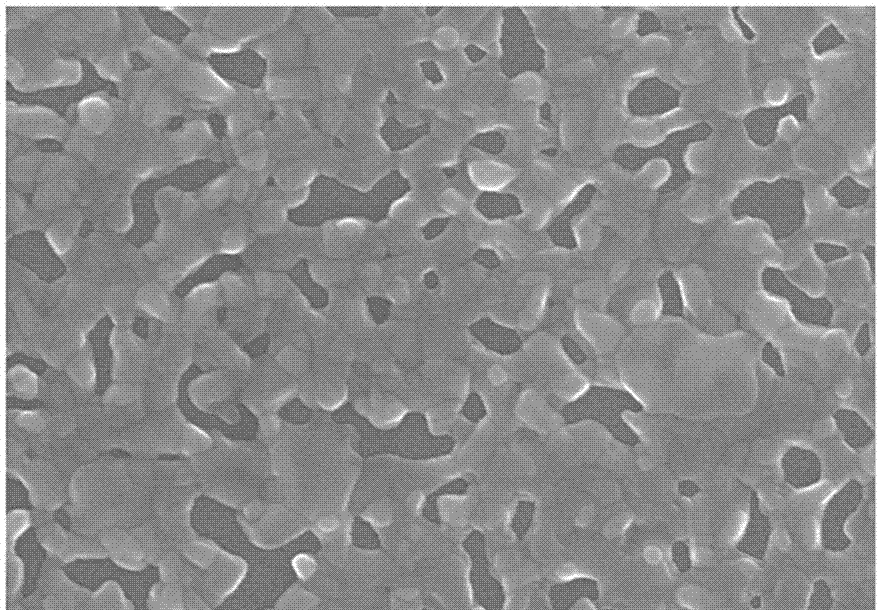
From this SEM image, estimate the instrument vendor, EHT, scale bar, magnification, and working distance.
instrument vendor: Hitachi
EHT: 15 kV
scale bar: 1000 nm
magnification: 30 K X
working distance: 11.4 mm